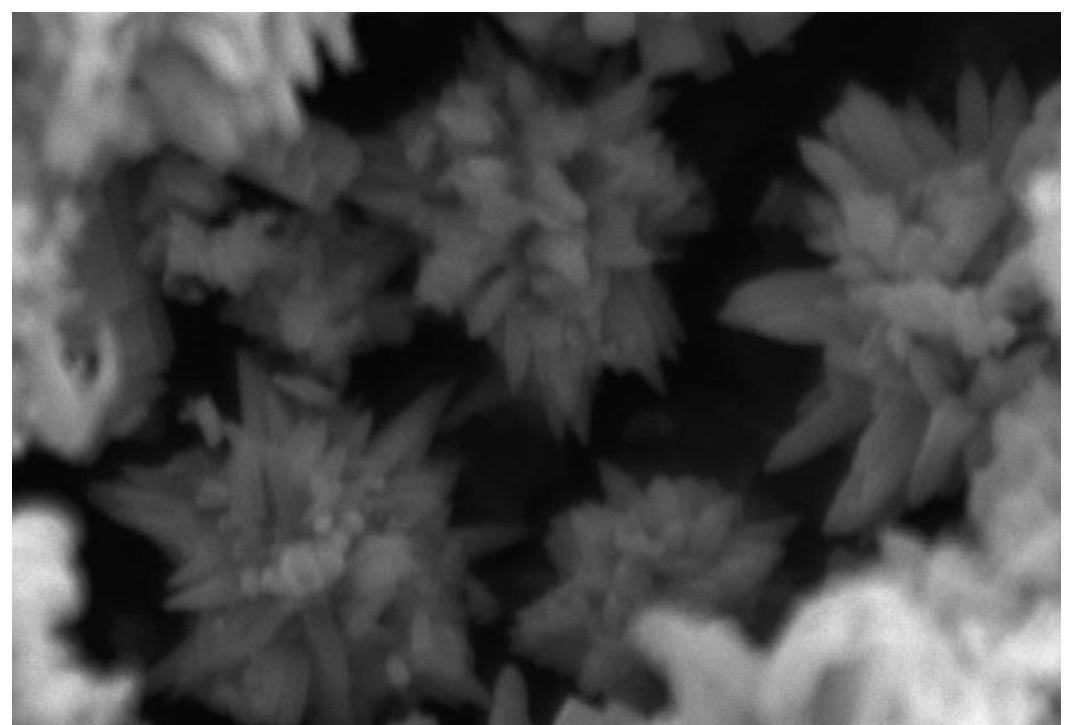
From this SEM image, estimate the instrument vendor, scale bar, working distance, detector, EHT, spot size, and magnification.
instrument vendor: JEOL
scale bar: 1000 nm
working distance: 14 mm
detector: SEI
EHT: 15 kV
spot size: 30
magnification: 10 K X